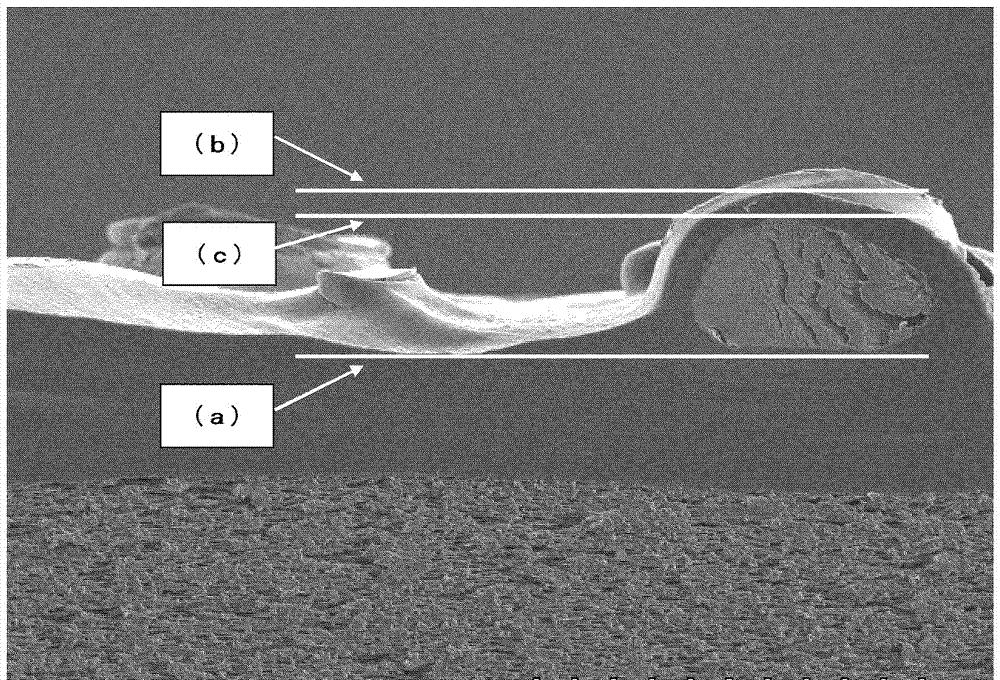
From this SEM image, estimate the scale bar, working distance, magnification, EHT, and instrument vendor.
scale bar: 50000 nm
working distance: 14.9 mm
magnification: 1 K X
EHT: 3 kV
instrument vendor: Hitachi